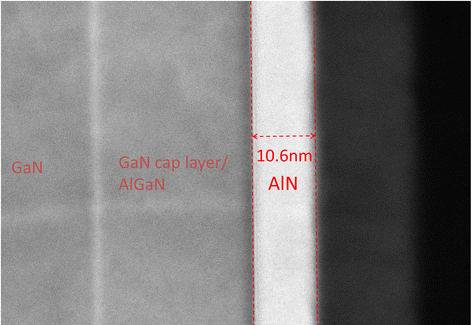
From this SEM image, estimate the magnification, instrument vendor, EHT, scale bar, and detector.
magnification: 1500 K X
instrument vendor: Hitachi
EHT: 200 kV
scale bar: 20 nm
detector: TE